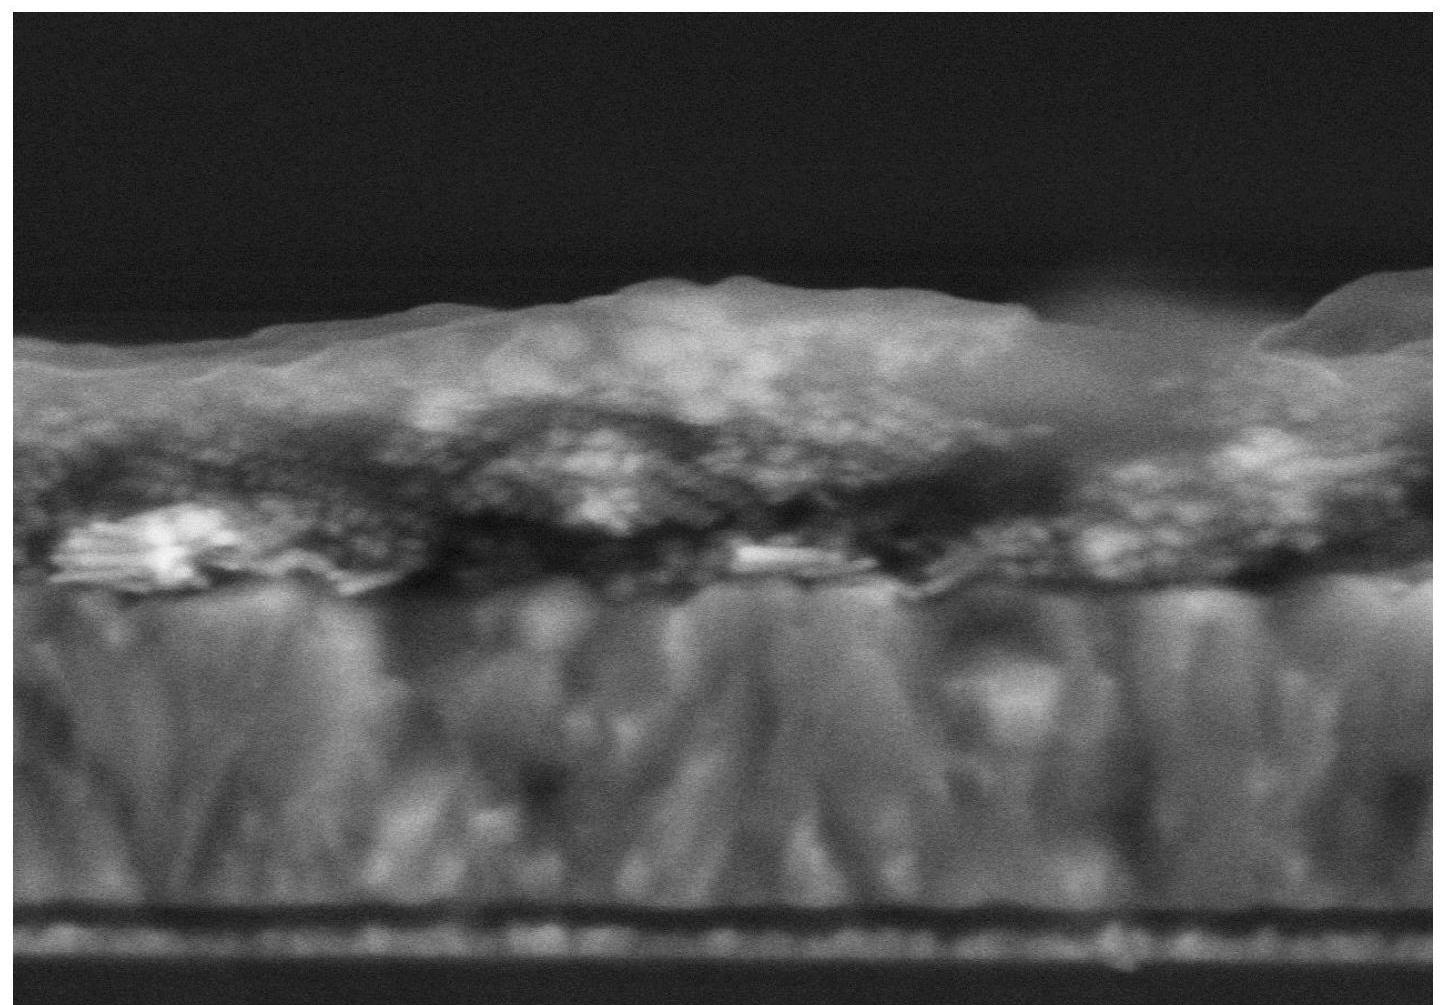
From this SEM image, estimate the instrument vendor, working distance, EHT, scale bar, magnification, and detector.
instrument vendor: Hitachi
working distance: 11 mm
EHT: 5 kV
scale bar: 500 nm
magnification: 110 K X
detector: SE(M)